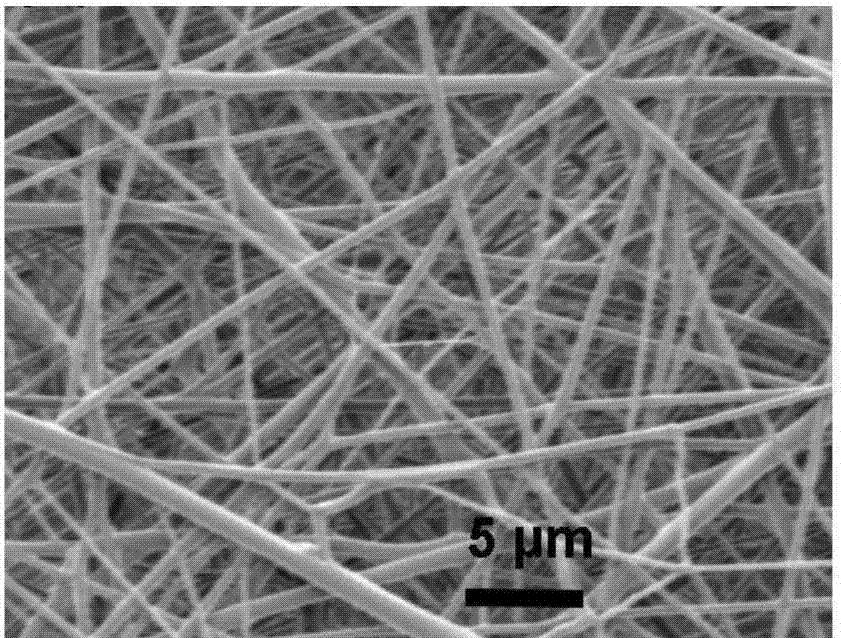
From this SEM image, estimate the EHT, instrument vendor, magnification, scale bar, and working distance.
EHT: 2 kV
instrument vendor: FEI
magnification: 10.404 K X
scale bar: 10000 nm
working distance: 35.9 mm